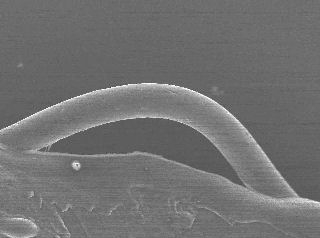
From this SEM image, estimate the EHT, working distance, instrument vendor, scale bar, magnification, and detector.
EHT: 10 kV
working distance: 10.2 mm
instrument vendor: JEOL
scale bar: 10000 nm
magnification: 0.7 K X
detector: SEI